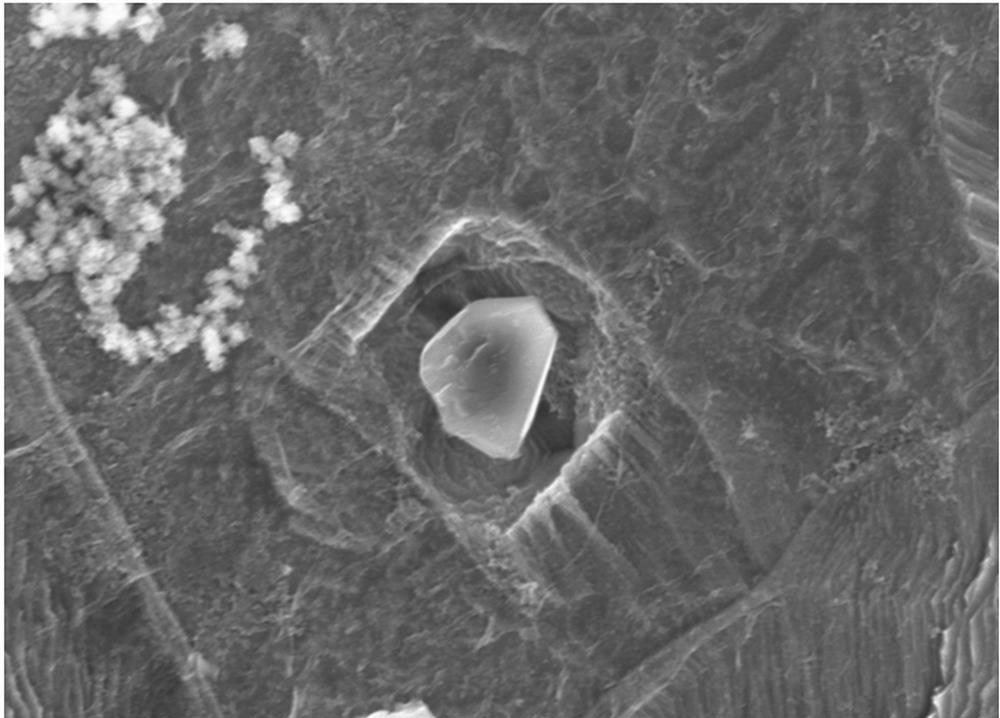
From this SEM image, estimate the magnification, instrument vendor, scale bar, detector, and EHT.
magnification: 5 K X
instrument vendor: Hitachi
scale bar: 10000 nm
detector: SE(M)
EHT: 15 kV